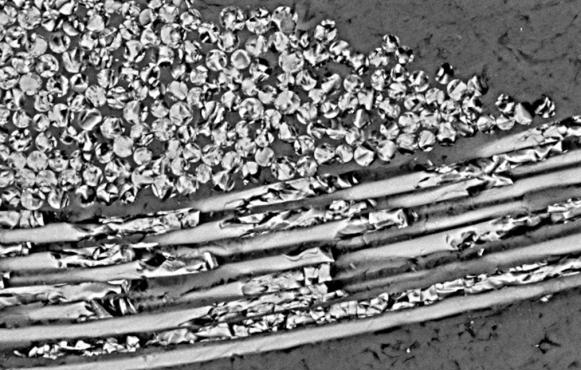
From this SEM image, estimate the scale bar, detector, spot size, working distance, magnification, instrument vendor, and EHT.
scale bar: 50000 nm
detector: BSE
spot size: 5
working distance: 10.4 mm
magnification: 0.35 K X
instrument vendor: FEI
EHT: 20 kV